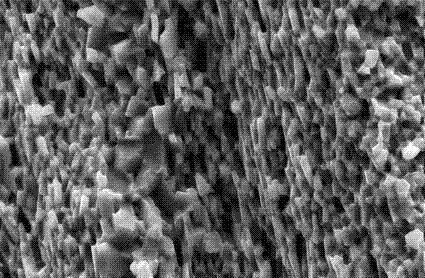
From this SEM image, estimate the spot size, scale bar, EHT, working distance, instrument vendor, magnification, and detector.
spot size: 4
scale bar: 2000 nm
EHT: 15 kV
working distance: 7.6 mm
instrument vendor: FEI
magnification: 20 K X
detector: SE